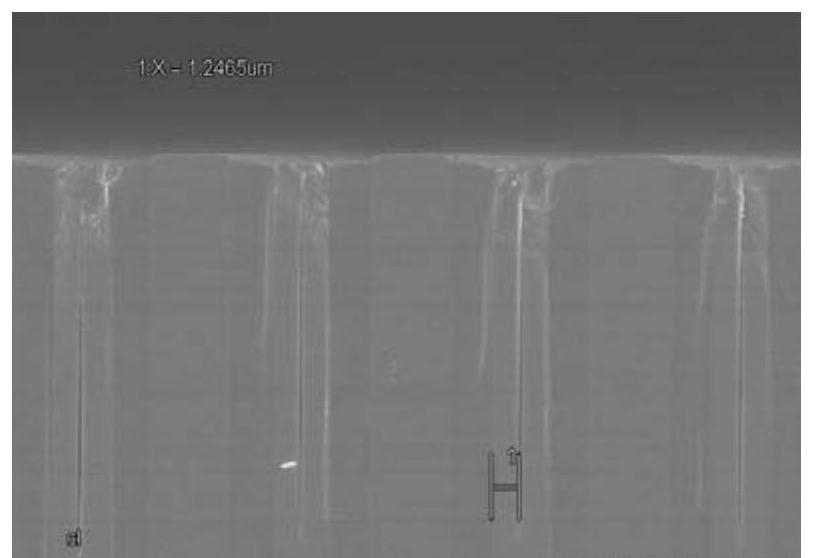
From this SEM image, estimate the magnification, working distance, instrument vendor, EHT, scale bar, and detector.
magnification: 3.5 K X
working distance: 11.6 mm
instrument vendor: Hitachi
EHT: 5 kV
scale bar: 10000 nm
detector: SE(M)